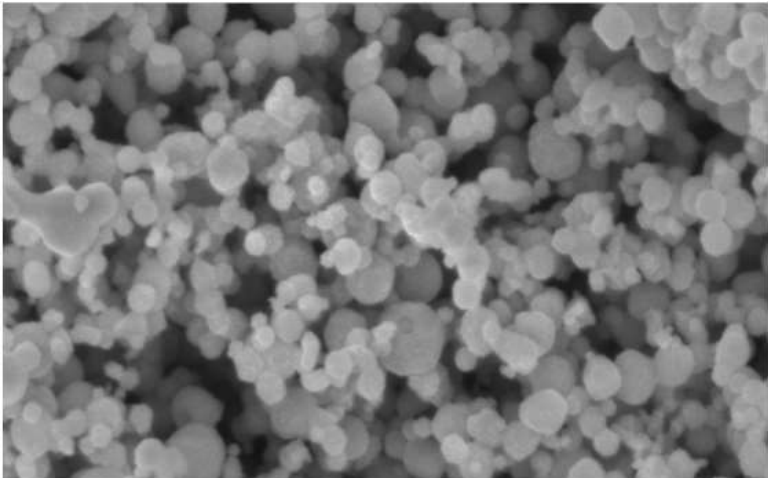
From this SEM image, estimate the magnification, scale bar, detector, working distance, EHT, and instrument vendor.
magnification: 50 K X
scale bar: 200 nm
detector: InLens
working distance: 5.9 mm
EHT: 5 kV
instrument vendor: Zeiss